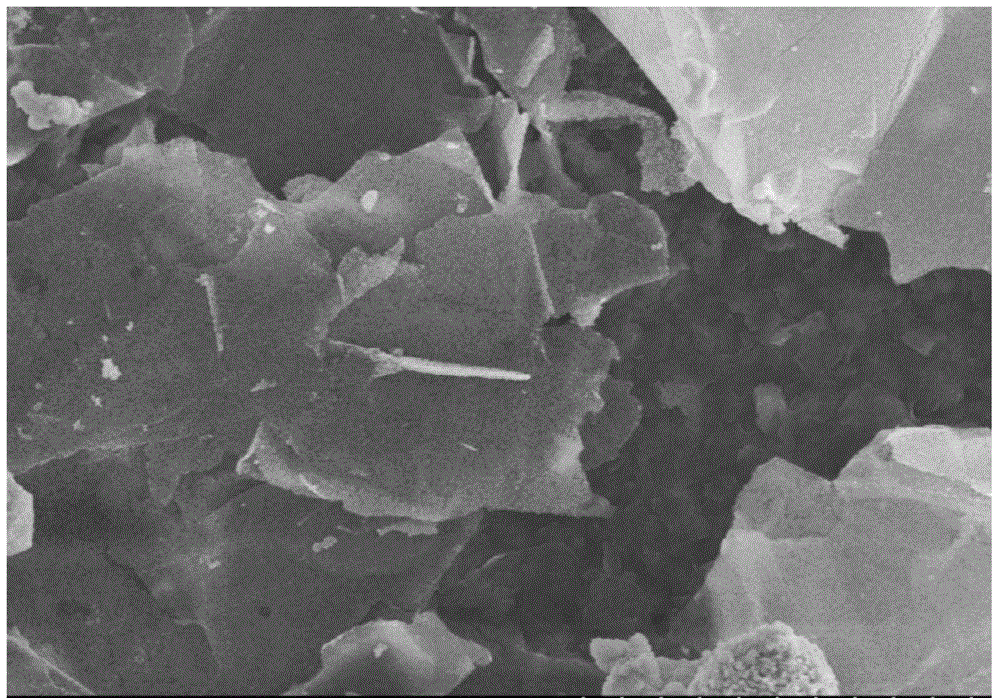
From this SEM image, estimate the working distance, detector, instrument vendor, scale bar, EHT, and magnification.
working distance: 9.2 mm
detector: SE(M)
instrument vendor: Hitachi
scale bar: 5000 nm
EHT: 5 kV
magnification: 10 K X